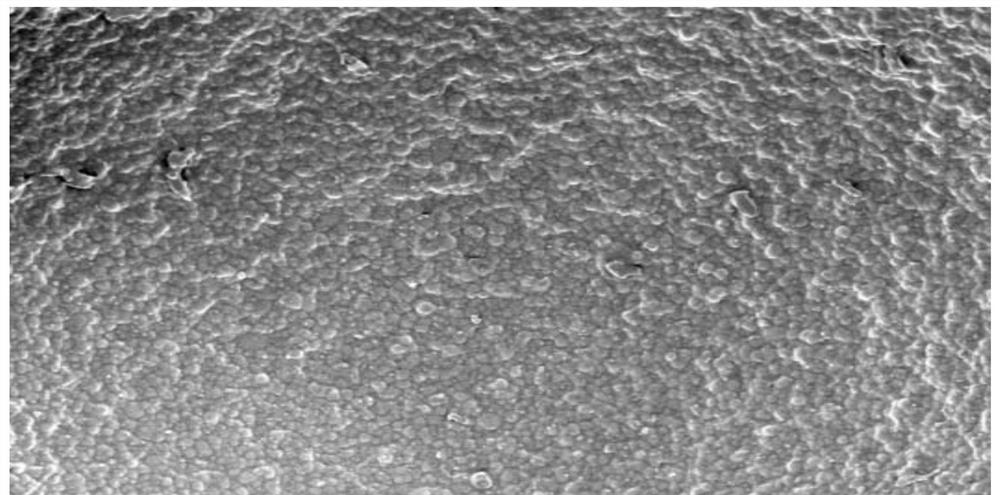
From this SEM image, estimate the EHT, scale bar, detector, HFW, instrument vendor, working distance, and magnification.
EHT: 2 kV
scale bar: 10000 nm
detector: ETD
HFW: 41.4 µm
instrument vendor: FEI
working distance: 8.9 mm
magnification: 10 K X